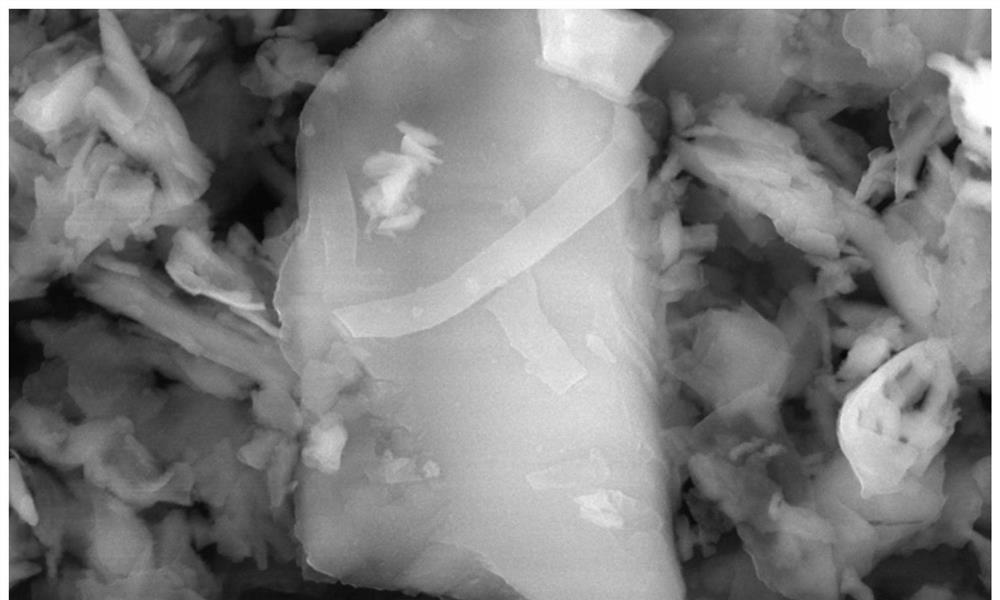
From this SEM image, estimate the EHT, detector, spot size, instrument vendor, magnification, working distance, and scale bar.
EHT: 15 kV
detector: TLD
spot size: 3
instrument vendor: FEI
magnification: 100 K X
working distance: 5 mm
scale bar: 1000 nm